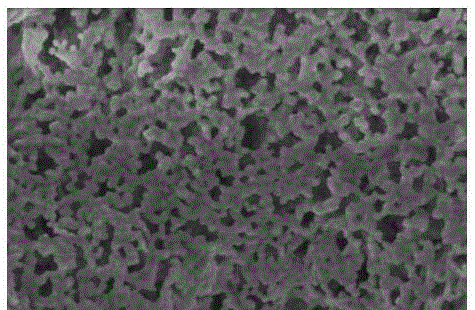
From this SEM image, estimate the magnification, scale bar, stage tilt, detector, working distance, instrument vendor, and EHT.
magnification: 500 K X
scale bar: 200 nm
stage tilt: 0°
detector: TLD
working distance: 3 mm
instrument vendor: FEI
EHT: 1 kV